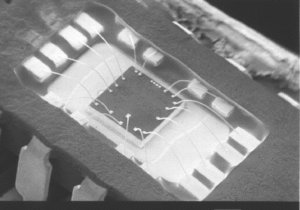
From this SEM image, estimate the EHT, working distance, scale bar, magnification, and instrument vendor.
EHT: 2 kV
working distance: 47 mm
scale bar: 1000000 nm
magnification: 0.01 K X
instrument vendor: JEOL